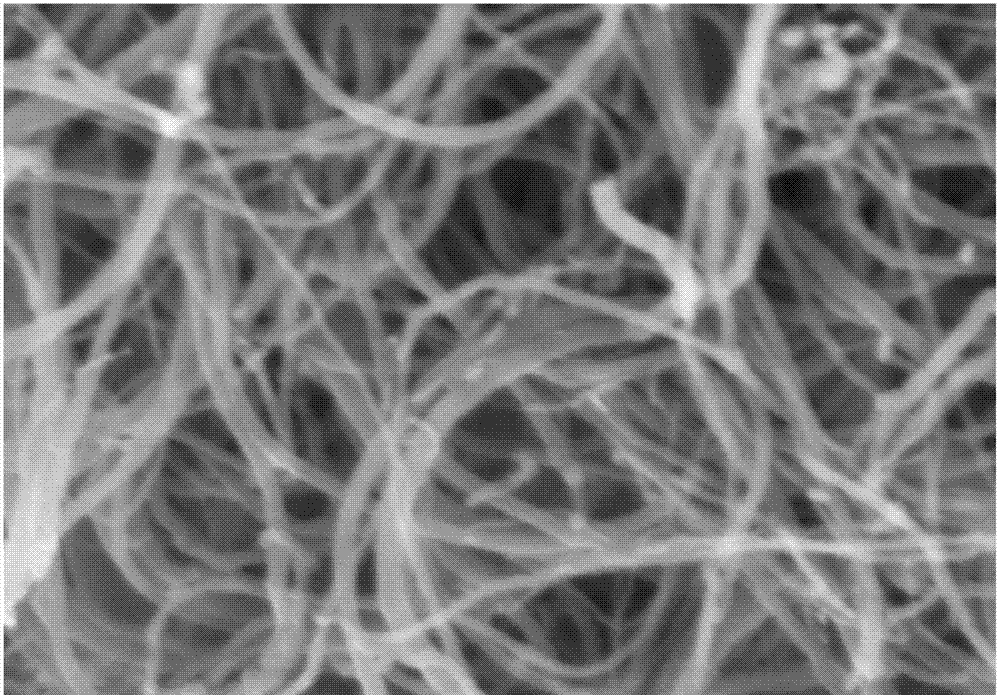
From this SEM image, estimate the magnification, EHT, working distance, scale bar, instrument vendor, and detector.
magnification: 70 K X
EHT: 3 kV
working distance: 8.5 mm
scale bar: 500 nm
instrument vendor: Hitachi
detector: SE(M)